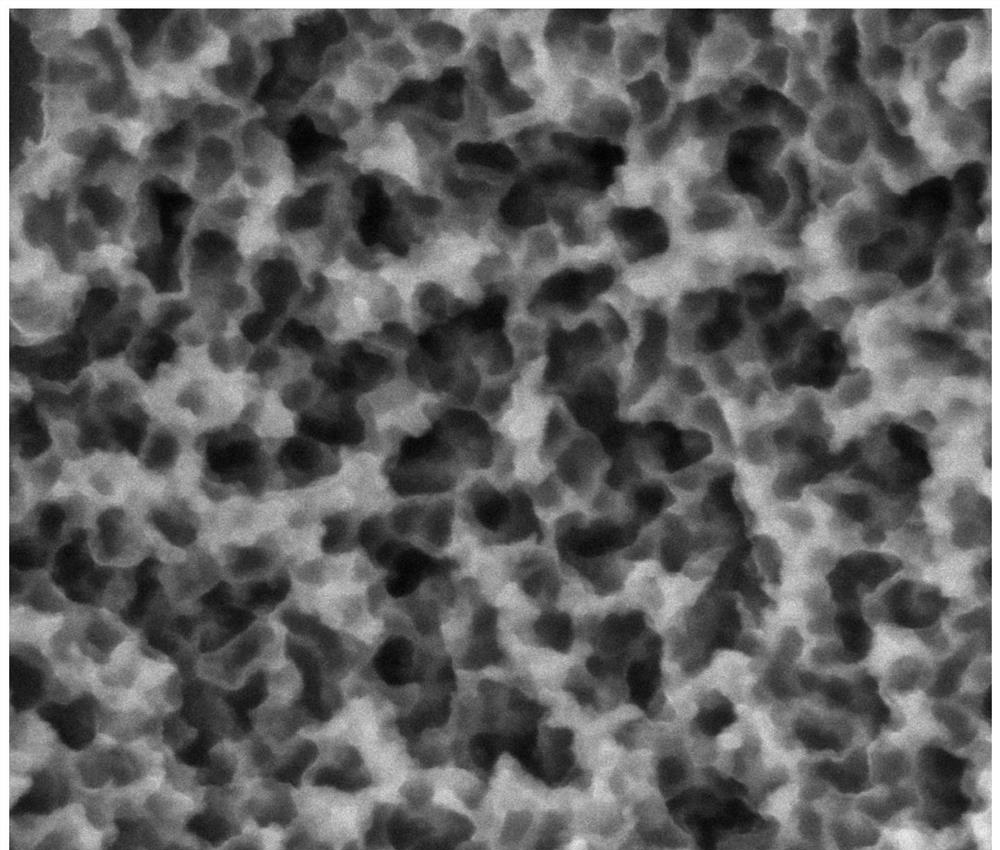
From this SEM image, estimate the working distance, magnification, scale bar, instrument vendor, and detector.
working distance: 9.8 mm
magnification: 50 K X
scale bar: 2000 nm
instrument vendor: FEI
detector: SE